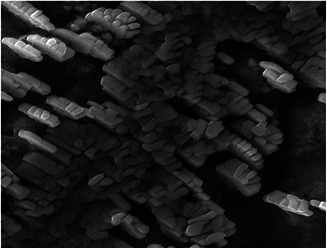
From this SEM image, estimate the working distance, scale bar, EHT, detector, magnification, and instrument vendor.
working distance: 15.19 mm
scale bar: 5000 nm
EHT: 20 kV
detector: SE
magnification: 10 K X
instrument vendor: Tescan